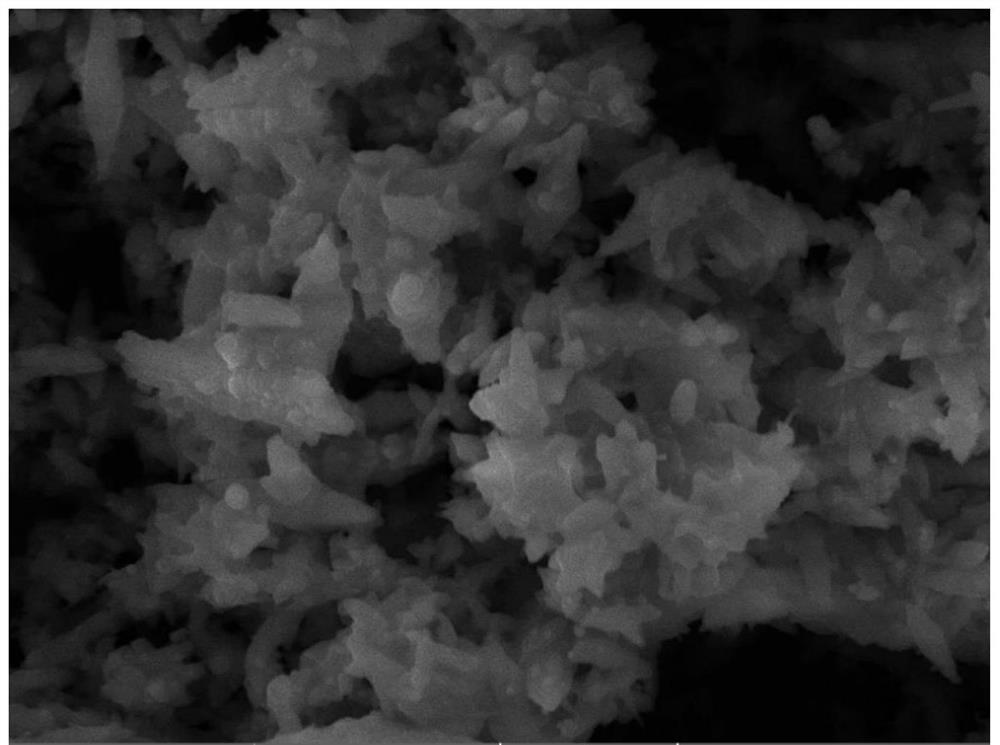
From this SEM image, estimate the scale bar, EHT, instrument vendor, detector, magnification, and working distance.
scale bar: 10000 nm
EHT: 20 kV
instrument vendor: Tescan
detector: SE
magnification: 5 K X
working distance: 10.08 mm